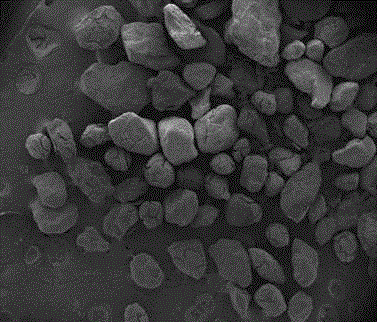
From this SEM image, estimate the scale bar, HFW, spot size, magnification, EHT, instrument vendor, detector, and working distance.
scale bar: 500000 nm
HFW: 2980 µm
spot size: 2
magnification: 0.05 K X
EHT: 10 kV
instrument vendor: FEI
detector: ETD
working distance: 5.6 mm